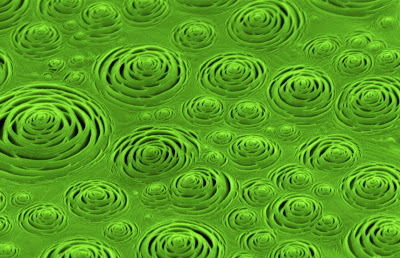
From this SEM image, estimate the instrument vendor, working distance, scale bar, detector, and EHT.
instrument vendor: Zeiss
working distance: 3 mm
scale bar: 1000 nm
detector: InLens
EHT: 3 kV